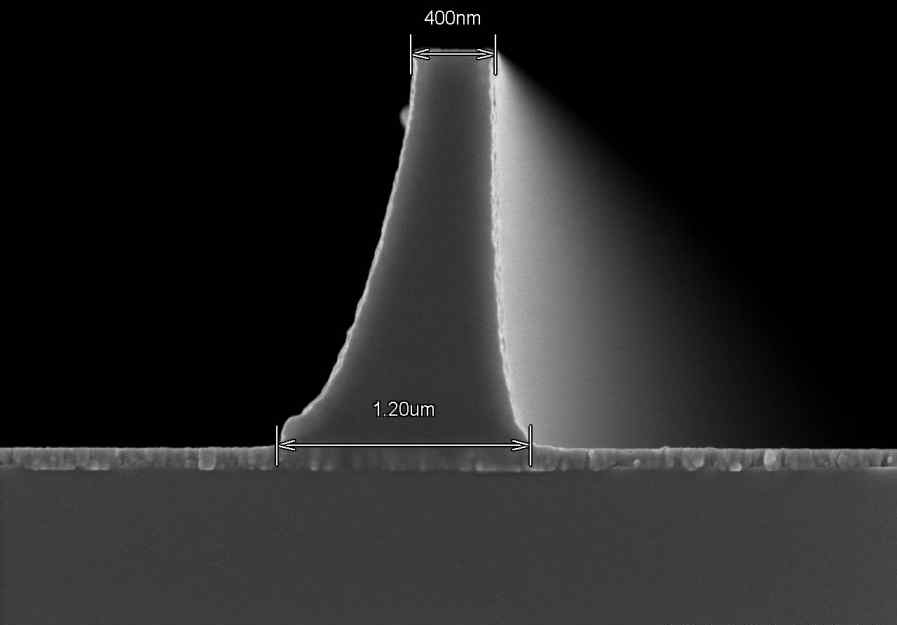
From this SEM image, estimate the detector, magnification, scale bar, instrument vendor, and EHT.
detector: SE(U,LA0)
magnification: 30 K X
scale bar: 1000 nm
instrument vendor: Hitachi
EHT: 3 kV